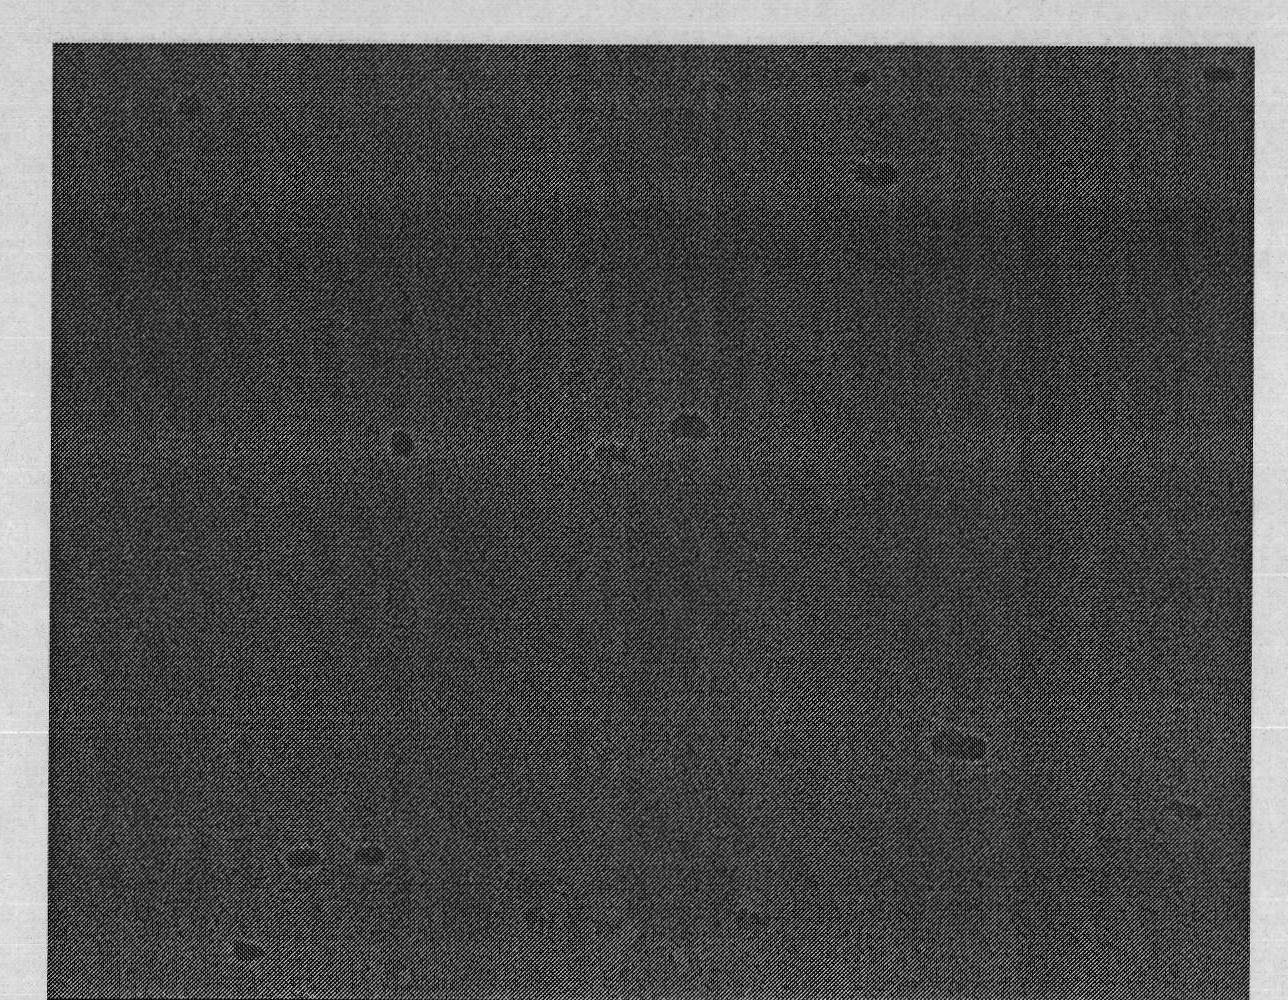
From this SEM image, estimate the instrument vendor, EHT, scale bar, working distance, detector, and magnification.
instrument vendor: Hitachi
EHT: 10 kV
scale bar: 1000 nm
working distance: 13.4 mm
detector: SE(M)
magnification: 30 K X